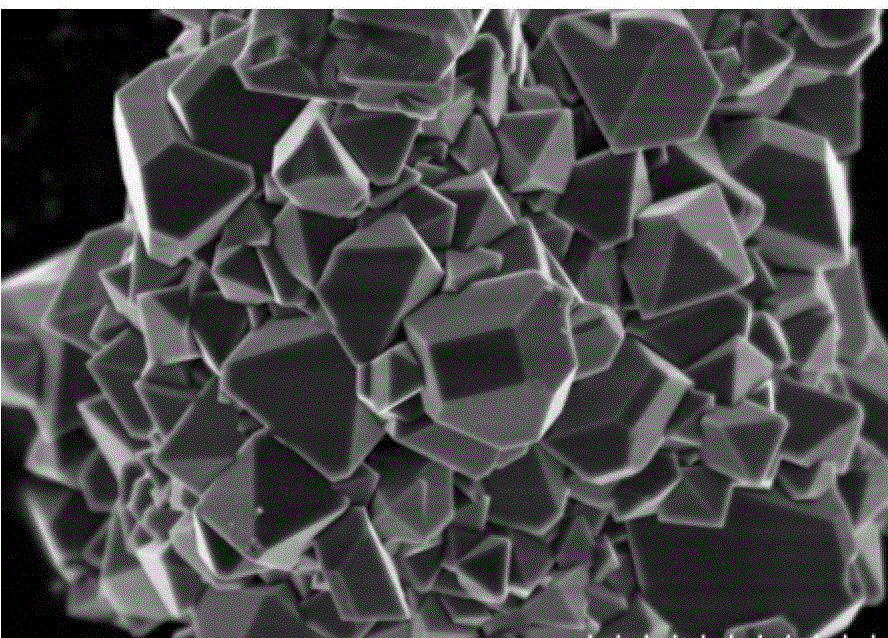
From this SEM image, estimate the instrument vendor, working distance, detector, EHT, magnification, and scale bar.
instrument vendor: Hitachi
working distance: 8.8 mm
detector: SE(M)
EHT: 3 kV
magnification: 20 K X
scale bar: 2000 nm